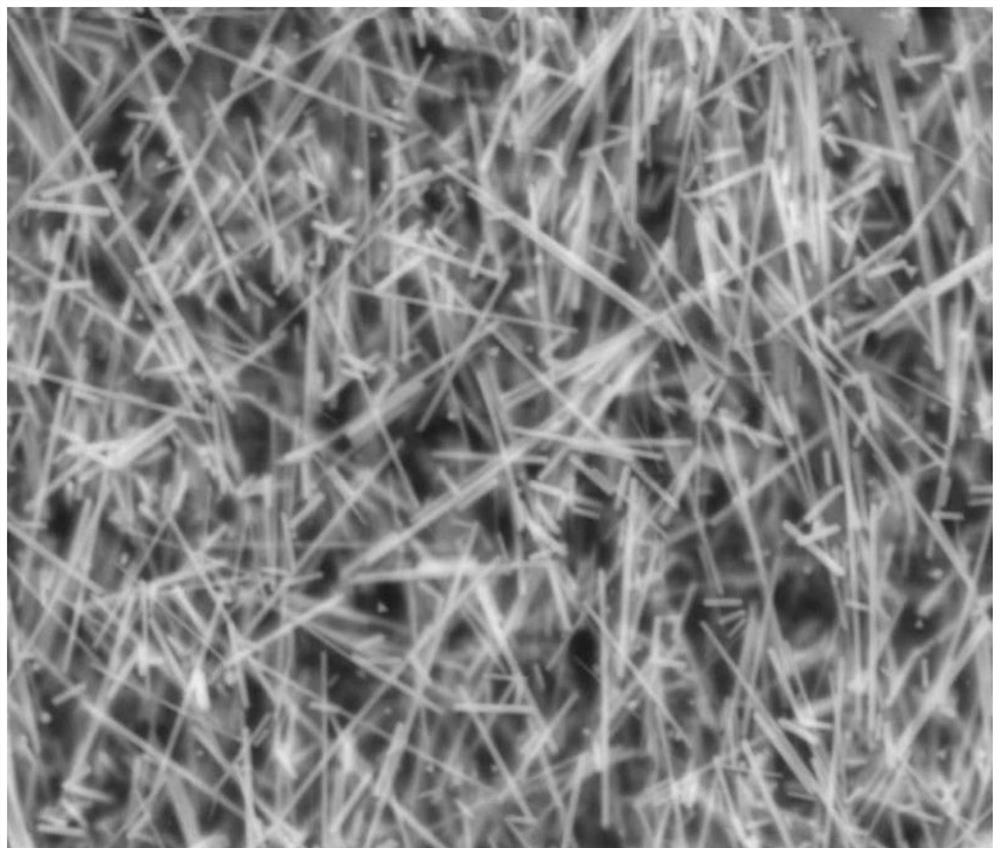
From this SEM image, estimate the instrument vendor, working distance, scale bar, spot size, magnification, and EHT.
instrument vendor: FEI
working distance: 9.7 mm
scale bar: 10000 nm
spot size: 4.5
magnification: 10 K X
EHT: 30 kV